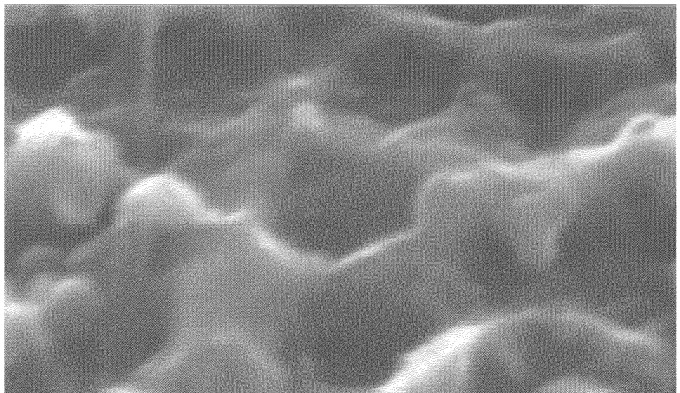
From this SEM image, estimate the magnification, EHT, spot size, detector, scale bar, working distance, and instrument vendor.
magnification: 120 K X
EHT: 30 kV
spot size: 3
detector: SE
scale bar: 200 nm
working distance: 5.4 mm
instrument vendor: FEI